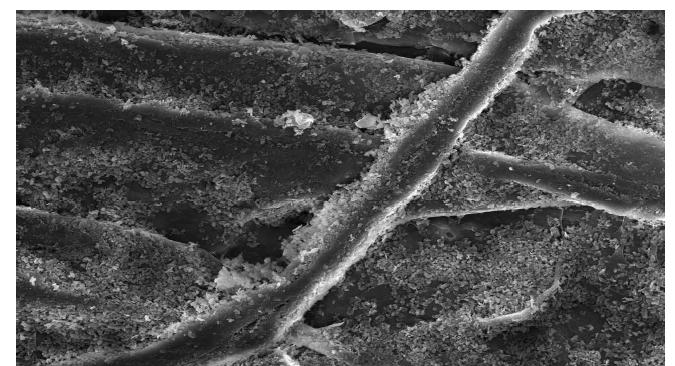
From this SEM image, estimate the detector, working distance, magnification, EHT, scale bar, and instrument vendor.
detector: SE(U)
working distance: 11.9 mm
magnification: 1 K X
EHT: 15 kV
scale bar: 50000 nm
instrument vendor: Hitachi